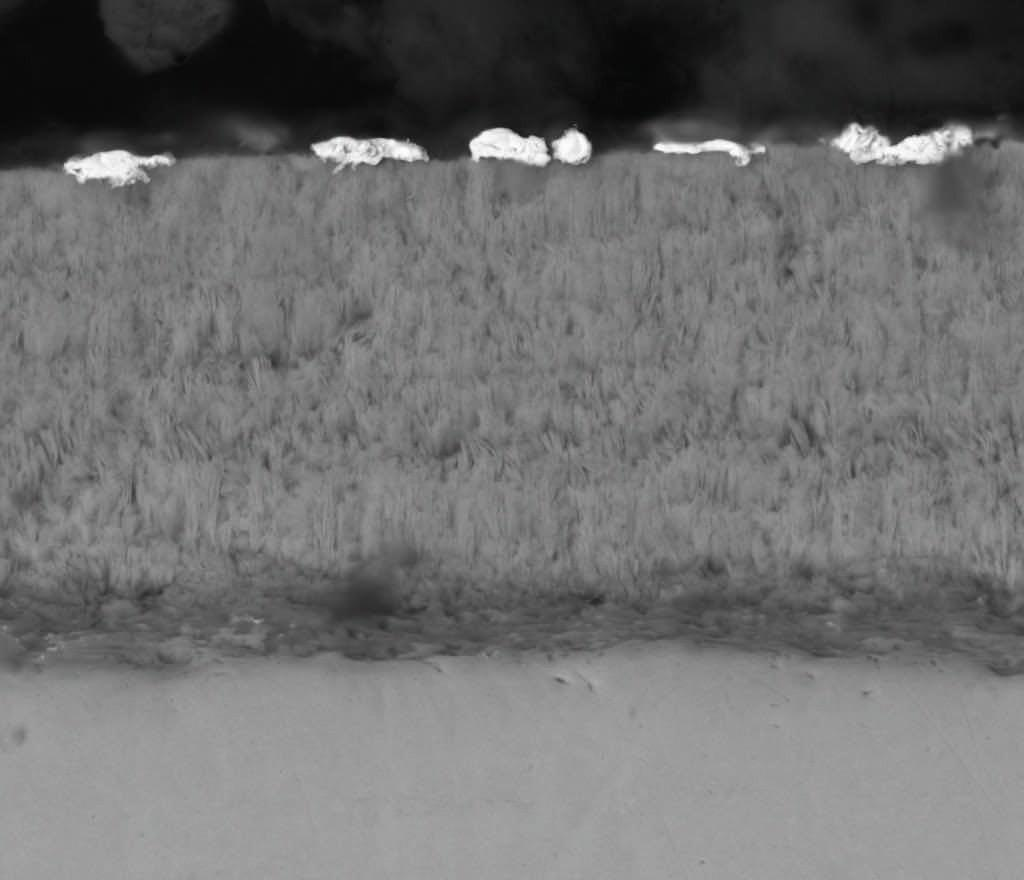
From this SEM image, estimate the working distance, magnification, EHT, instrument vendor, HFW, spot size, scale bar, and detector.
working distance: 7.1 mm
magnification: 10 K X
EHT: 15 kV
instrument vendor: FEI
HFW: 29.8 µm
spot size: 4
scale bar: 10000 nm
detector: DualBSD